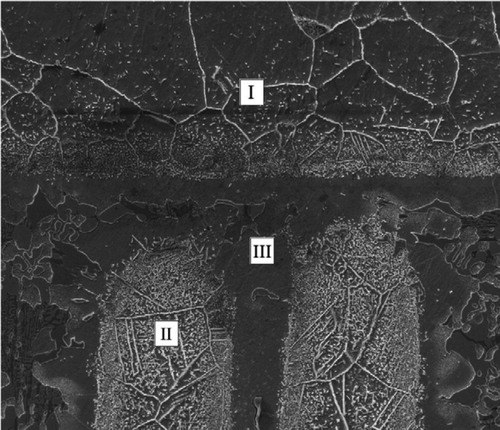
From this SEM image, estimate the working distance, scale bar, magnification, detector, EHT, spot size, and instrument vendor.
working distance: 12.1 mm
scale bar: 50000 nm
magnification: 0.5 K X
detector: ETD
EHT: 15 kV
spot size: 4.5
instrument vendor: FEI